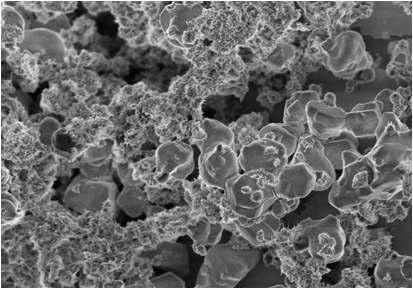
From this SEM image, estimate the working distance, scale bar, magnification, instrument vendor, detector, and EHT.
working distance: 3.4 mm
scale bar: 10000 nm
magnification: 3 K X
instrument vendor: Hitachi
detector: SE(M)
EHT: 3 kV